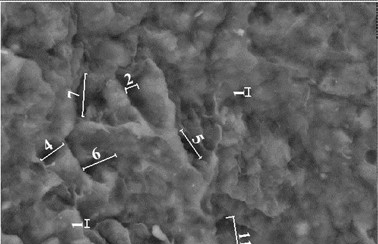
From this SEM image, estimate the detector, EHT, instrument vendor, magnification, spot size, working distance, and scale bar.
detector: BSE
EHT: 20 kV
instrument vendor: FEI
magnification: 4 K X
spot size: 4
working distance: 10.1 mm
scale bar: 10000 nm